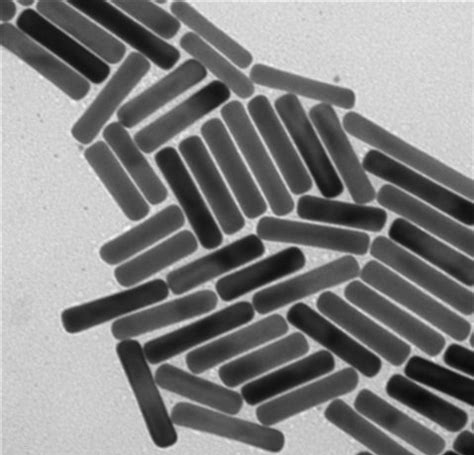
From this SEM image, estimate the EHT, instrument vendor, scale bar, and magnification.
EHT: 100 kV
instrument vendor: other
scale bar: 20 nm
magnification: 73 K X